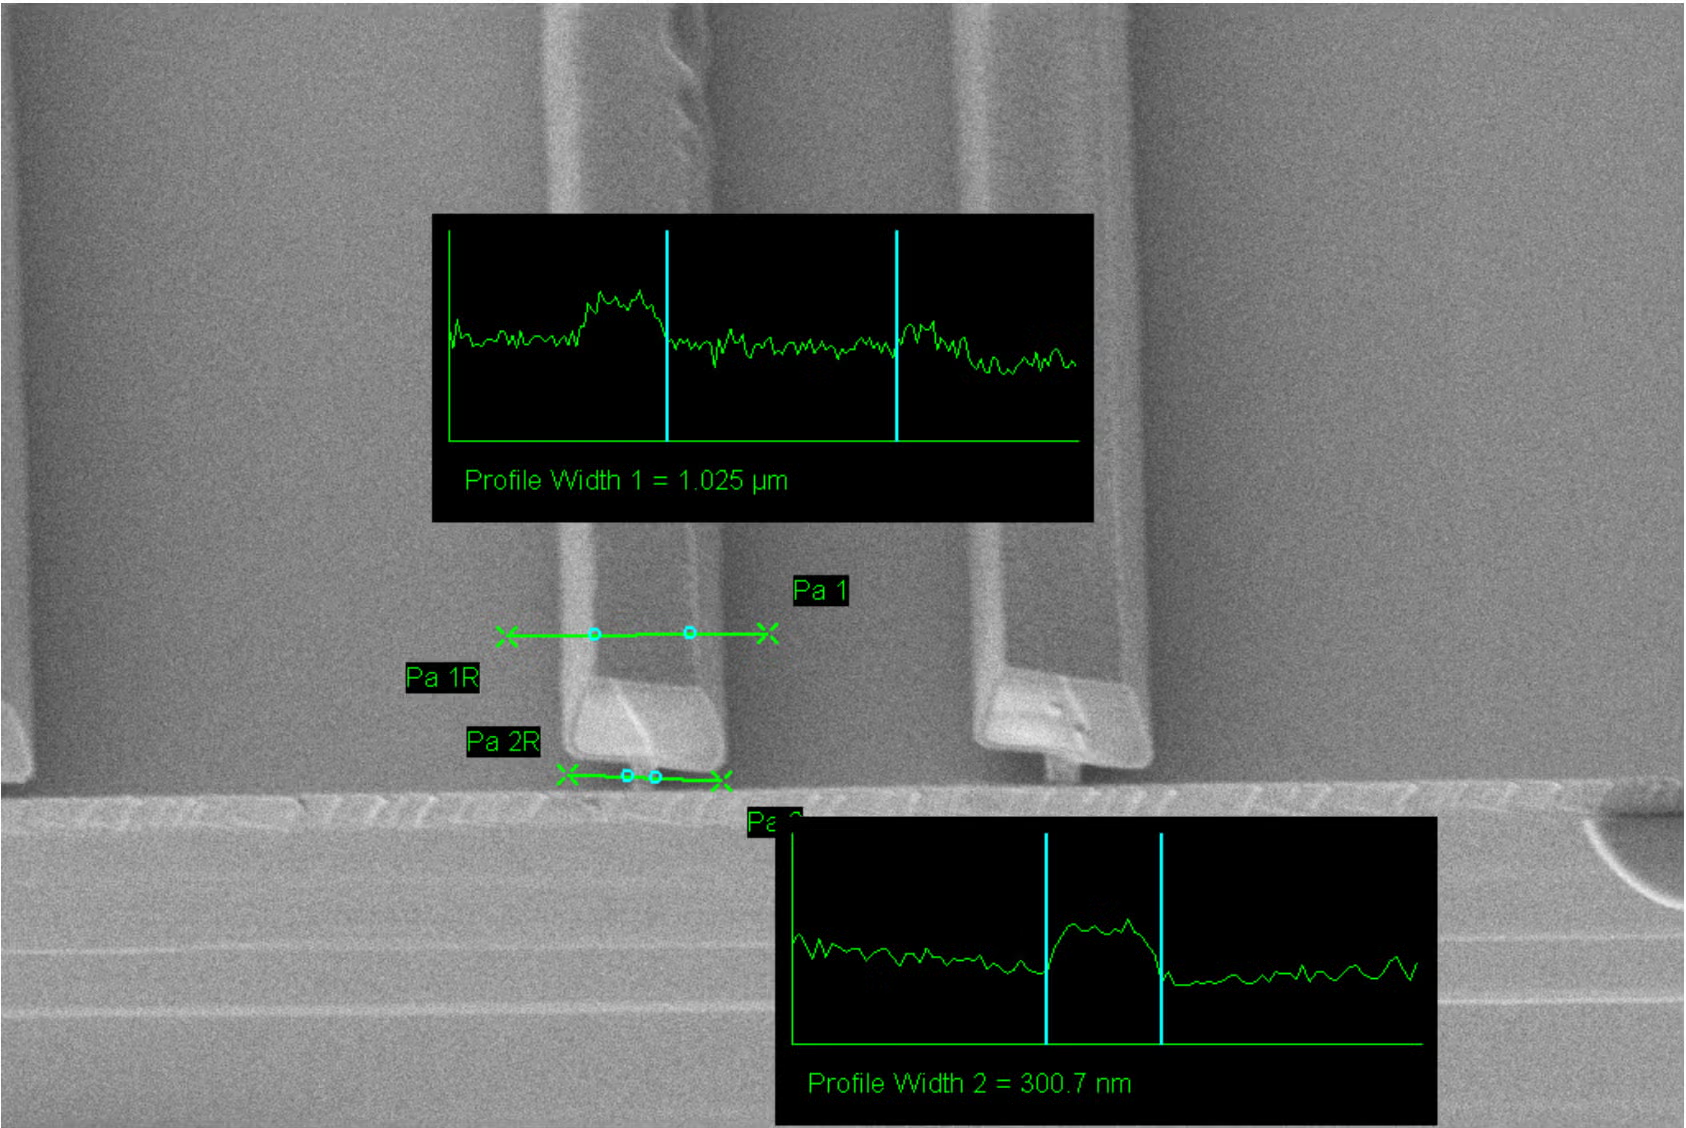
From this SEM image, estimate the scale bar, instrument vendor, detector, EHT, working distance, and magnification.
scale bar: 1000 nm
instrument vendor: Zeiss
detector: SE2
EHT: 2 kV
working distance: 7.9 mm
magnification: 6.32 K X